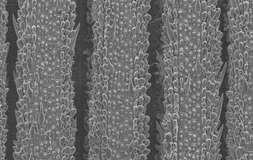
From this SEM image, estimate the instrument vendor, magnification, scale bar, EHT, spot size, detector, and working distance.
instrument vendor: FEI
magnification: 0.2 K X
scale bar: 100000 nm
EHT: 10 kV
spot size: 2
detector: SE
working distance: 10 mm